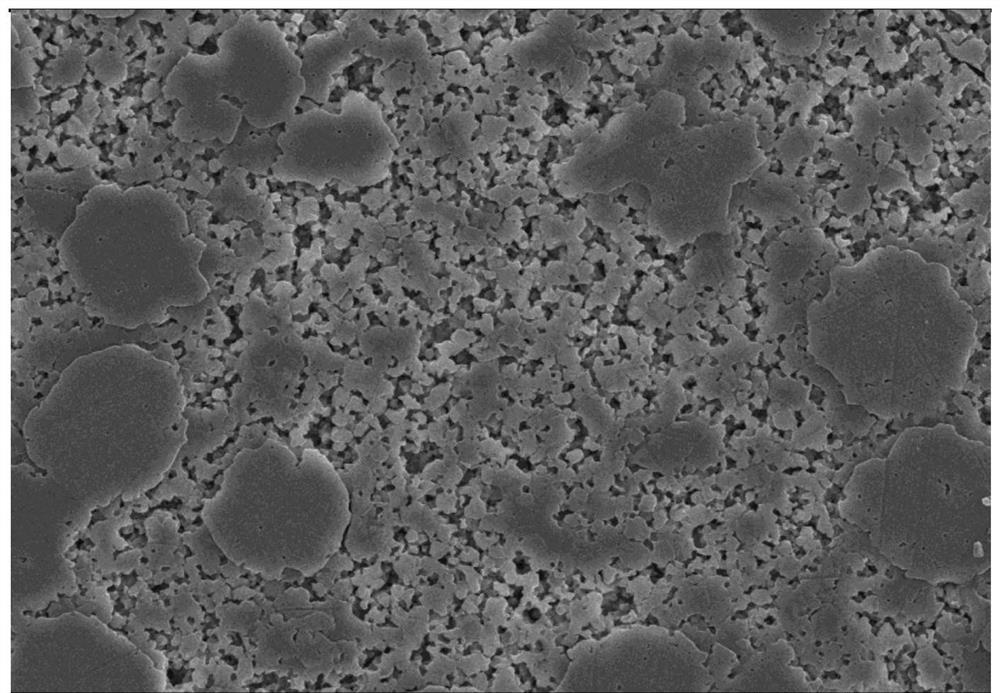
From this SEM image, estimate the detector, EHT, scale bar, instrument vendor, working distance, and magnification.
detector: SE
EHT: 15 kV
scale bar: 20000 nm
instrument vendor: Hitachi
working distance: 10.1 mm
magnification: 2 K X